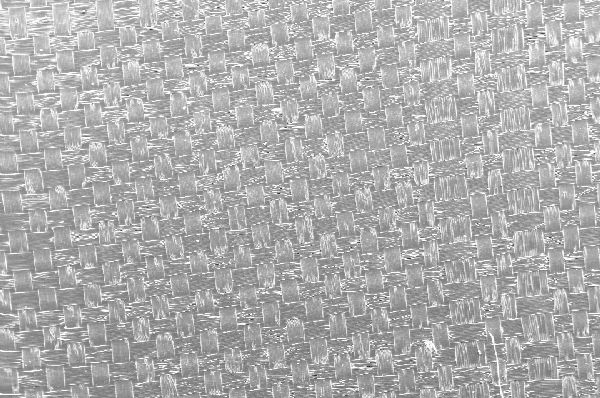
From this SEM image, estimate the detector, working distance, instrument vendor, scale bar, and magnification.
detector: SE1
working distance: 19 mm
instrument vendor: Zeiss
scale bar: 200000 nm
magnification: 0.036 K X